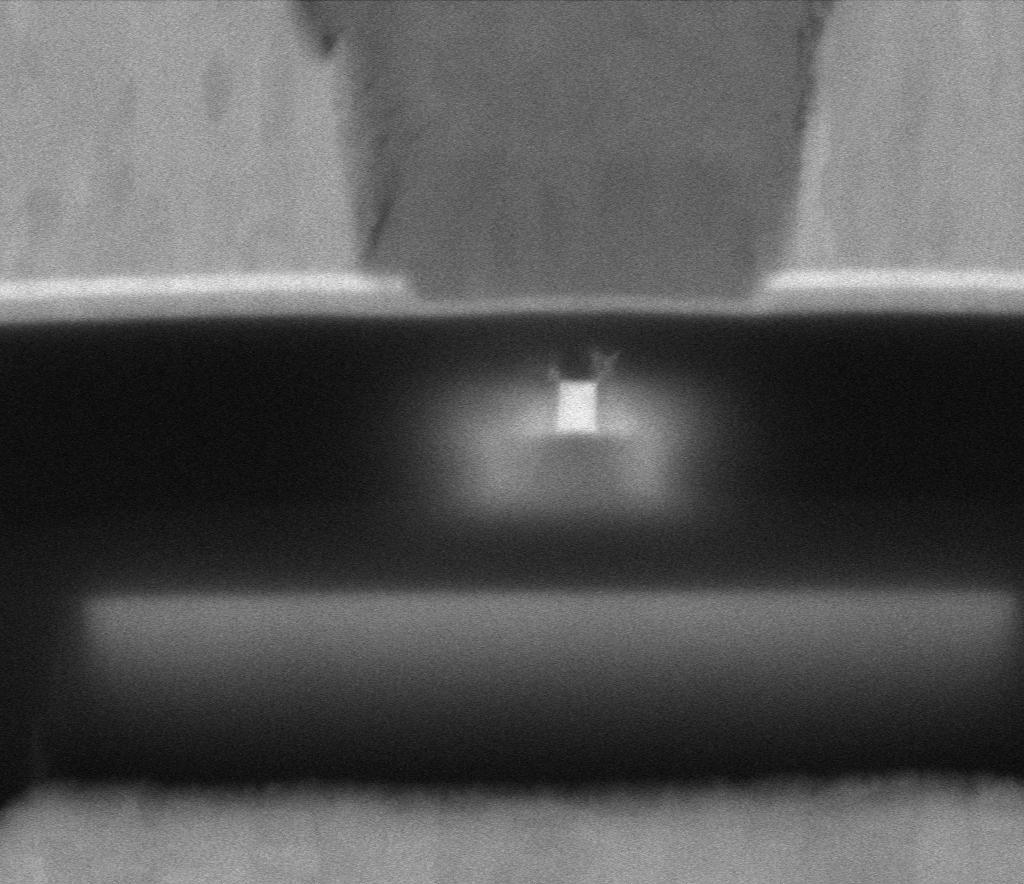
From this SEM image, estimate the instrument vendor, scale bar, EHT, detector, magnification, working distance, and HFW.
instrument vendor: Thermo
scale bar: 100 nm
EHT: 5 kV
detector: MD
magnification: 568.609 K X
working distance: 2 mm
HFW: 0.635 µm